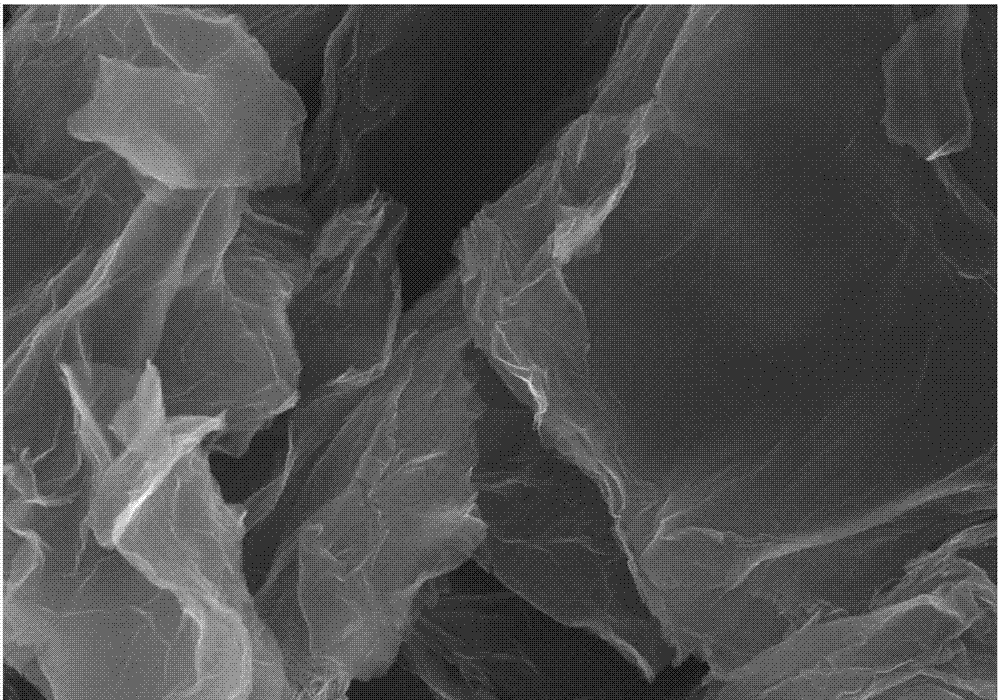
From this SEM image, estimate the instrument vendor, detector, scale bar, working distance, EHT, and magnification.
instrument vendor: Zeiss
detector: InlensDuo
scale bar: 1000 nm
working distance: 5.3 mm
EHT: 5 kV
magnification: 50 K X